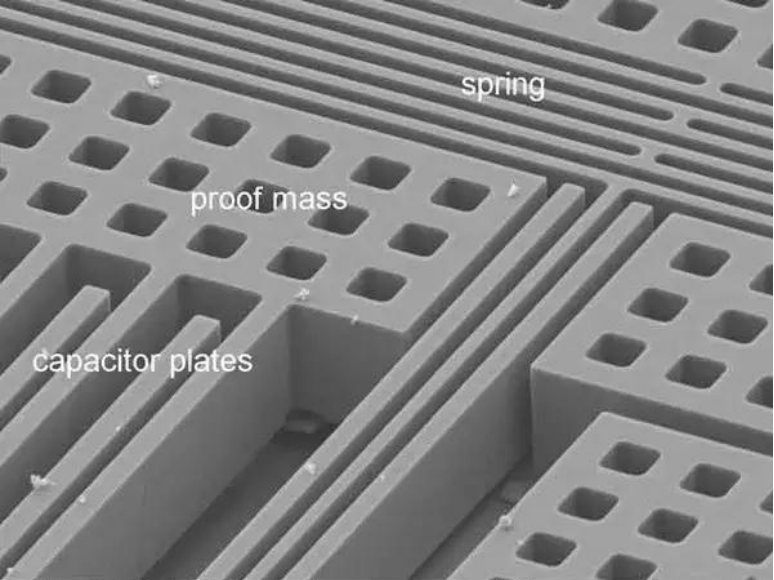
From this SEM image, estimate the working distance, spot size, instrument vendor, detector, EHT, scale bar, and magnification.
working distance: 14.6 mm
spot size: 3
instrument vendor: FEI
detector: SE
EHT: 10 kV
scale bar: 20000 nm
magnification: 1.5 K X